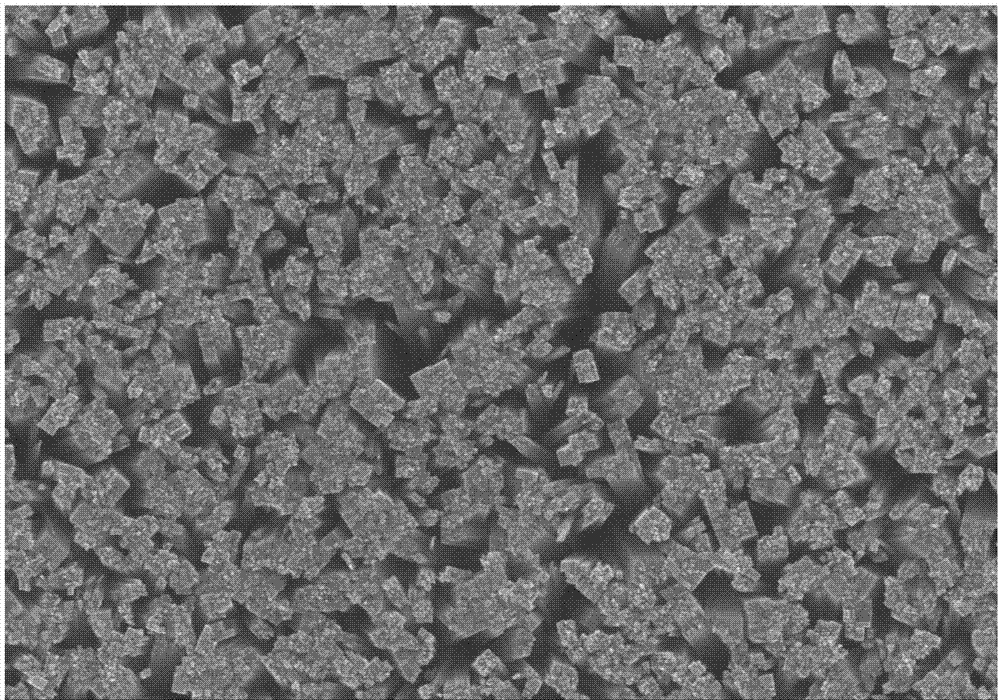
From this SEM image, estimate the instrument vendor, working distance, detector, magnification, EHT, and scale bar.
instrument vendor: Hitachi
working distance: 8.5 mm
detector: SE(M)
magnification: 20 K X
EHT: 20 kV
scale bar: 2000 nm